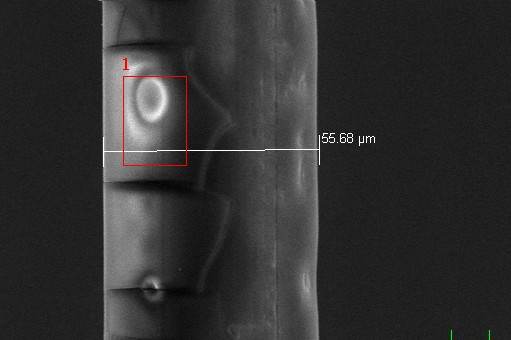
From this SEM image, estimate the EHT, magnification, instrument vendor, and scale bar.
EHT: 20 kV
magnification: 1 K X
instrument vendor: JEOL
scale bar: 10000 nm